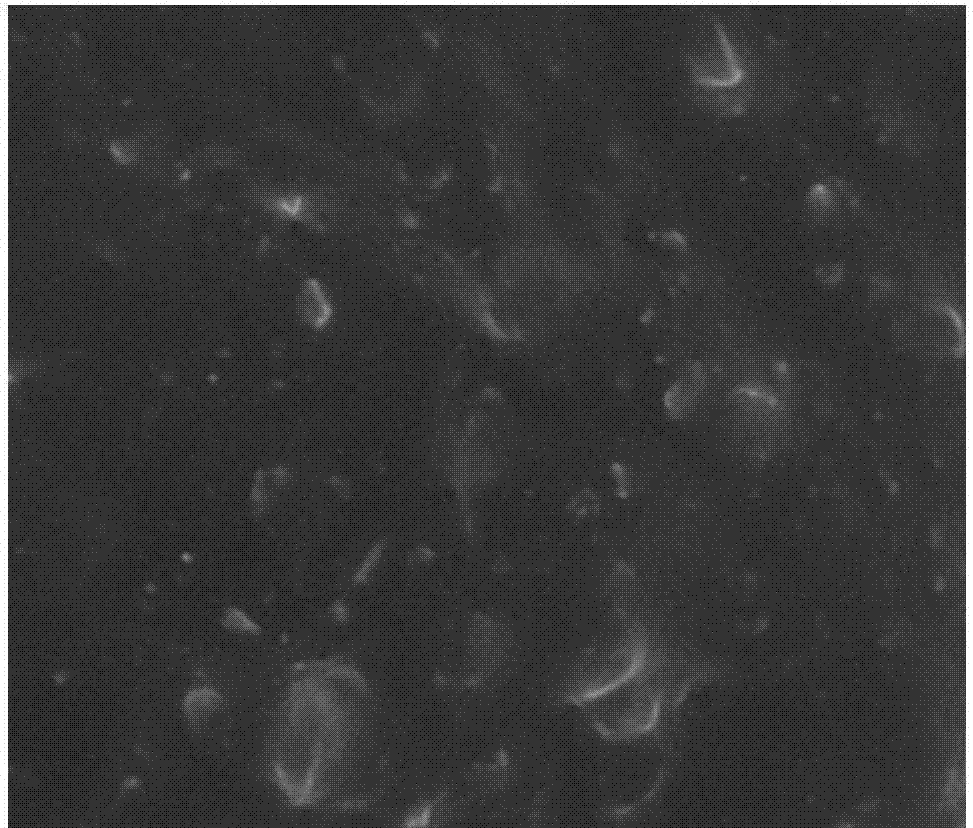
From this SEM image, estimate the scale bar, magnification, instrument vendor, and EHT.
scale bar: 1000 nm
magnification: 40 K X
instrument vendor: Tescan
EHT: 10 kV